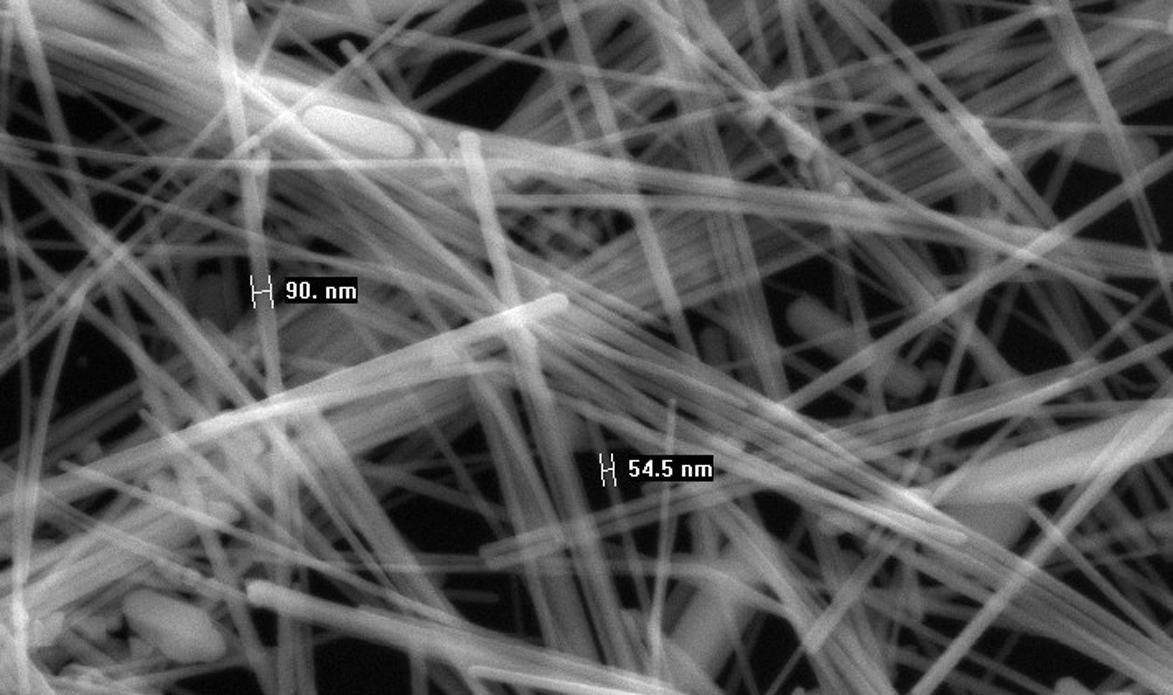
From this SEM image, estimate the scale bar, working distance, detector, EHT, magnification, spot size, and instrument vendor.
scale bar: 1000 nm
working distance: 6 mm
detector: SE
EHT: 25 kV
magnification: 50 K X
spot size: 4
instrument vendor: FEI